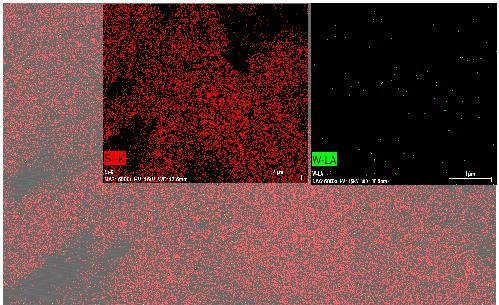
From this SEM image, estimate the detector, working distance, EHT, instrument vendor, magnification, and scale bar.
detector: SE BSE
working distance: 17.6 mm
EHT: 15 kV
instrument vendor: other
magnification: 6 K X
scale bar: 4000 nm